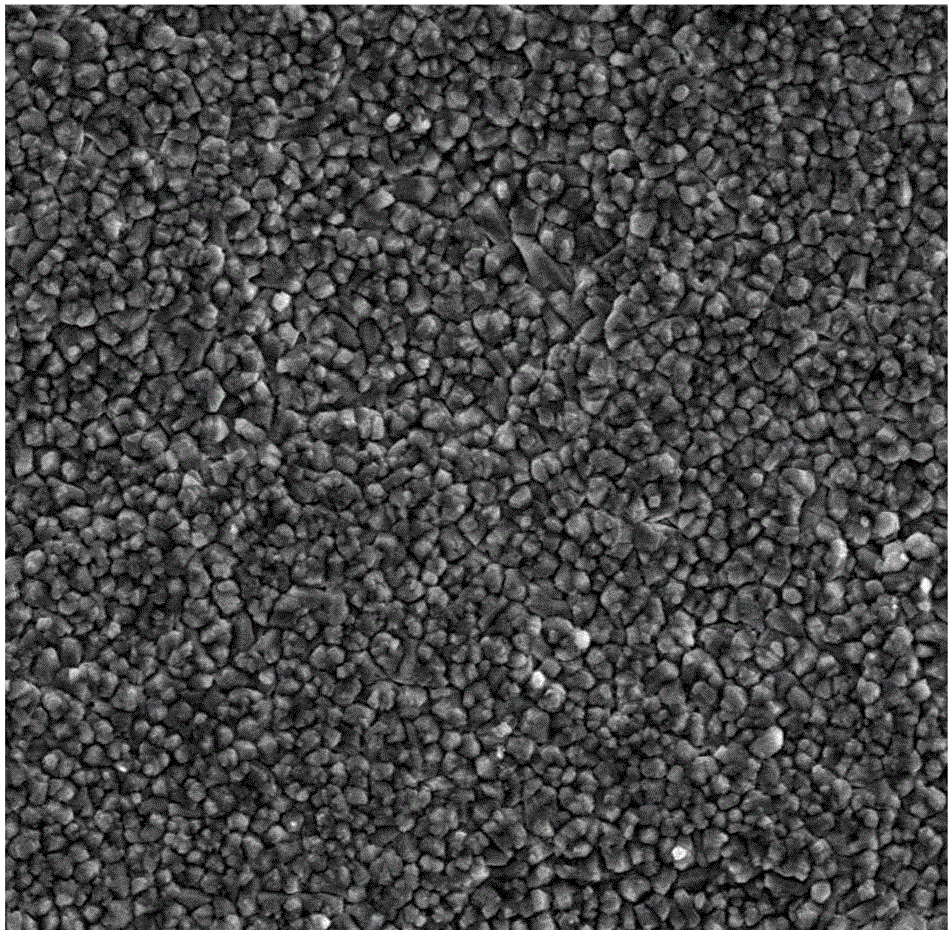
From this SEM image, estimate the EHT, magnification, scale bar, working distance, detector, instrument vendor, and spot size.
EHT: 20 kV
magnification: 1.6 K X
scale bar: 20000 nm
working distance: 12.2 mm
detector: SSD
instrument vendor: FEI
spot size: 4.5